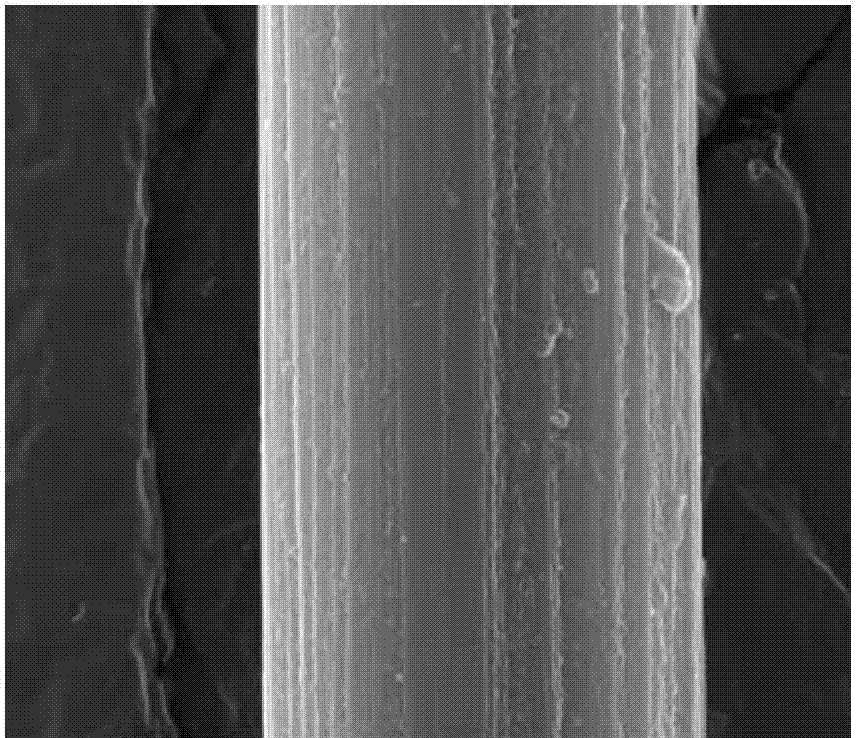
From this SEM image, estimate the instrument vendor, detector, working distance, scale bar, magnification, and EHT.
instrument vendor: FEI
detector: SE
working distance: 14 mm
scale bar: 5000 nm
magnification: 20 K X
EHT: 20 kV